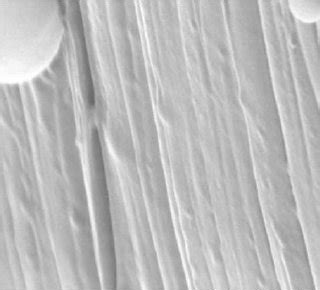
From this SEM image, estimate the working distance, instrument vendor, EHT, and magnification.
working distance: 19.9 mm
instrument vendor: Hitachi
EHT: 20 kV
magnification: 9 K X